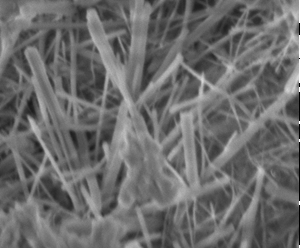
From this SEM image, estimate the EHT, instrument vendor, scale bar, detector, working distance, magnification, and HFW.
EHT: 5 kV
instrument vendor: FEI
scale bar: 5000 nm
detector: ETD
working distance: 9.3 mm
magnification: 15 K X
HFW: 9.95 µm